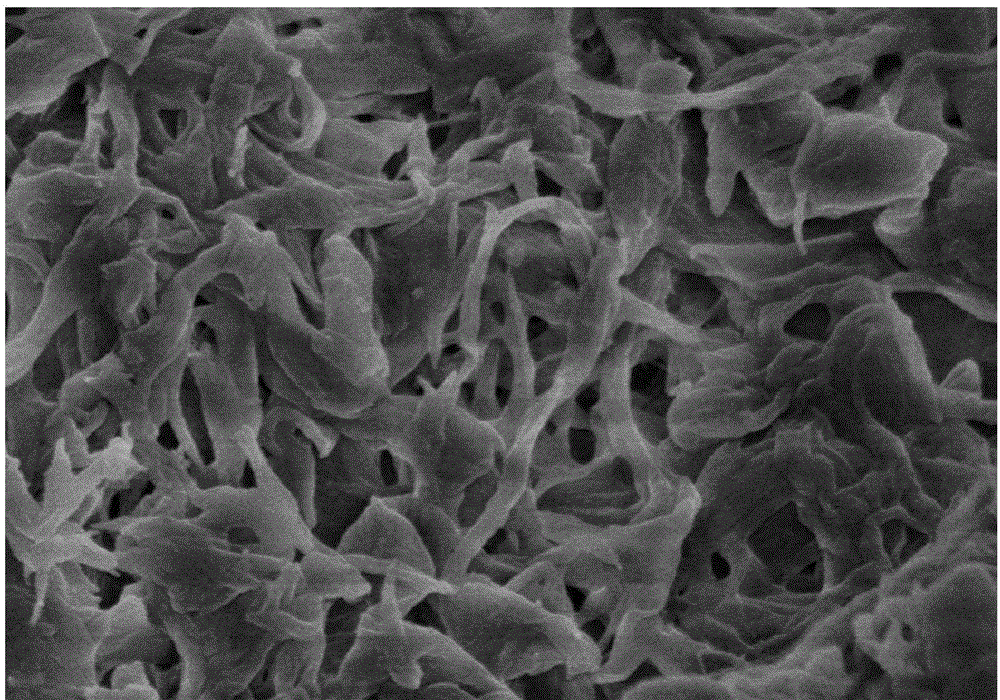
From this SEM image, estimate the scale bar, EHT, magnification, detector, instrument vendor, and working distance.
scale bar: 1000 nm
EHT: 3 kV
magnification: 30 K X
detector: SE(M)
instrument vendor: Hitachi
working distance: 10.4 mm